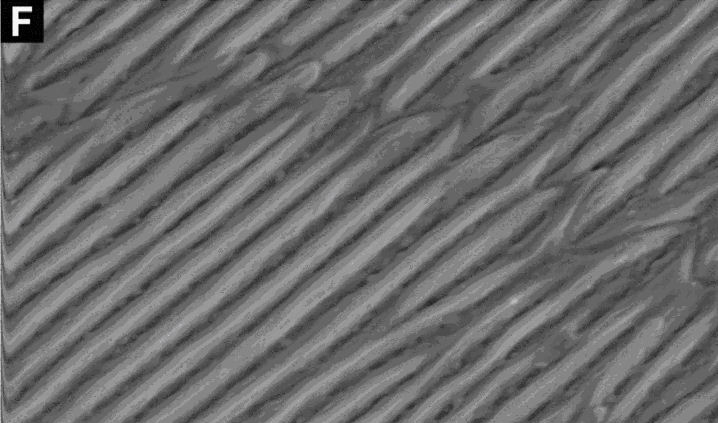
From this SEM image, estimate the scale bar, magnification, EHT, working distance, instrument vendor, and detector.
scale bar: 2000 nm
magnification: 10 K X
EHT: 10 kV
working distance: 32 mm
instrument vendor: FEI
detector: SE